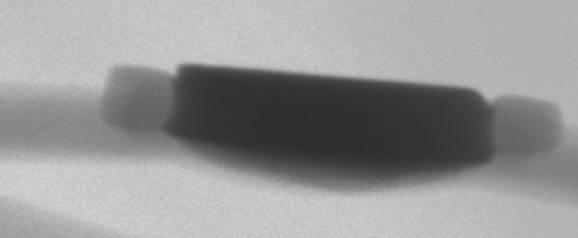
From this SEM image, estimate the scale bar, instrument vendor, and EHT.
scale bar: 500000 nm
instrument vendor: other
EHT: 100 kV